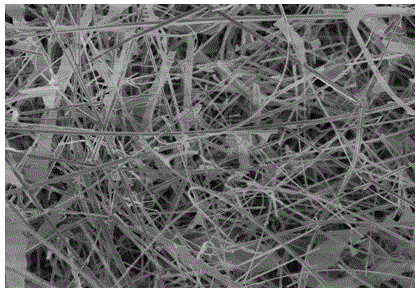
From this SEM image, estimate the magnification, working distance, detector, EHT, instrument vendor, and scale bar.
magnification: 3 K X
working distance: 9.5 mm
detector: SE(U)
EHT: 5 kV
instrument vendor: Hitachi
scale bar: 10000 nm